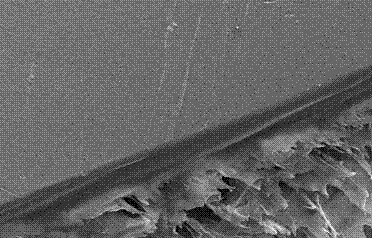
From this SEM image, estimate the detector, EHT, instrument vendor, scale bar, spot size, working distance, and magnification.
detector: SE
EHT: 25 kV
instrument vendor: FEI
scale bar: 100000 nm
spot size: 4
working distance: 6.6 mm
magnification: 0.5 K X